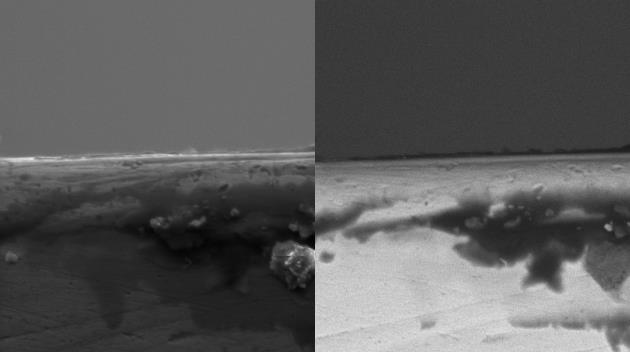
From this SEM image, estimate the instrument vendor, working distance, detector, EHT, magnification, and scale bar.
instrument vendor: Tescan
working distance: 17.38 mm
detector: SE, BSE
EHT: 10 kV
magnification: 5.44 K X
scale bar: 20000 nm